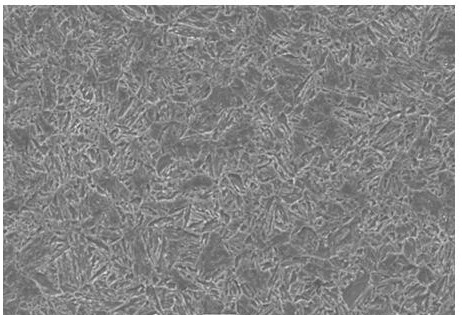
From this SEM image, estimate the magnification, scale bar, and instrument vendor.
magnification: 1 K X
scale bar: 10000 nm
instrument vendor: JEOL